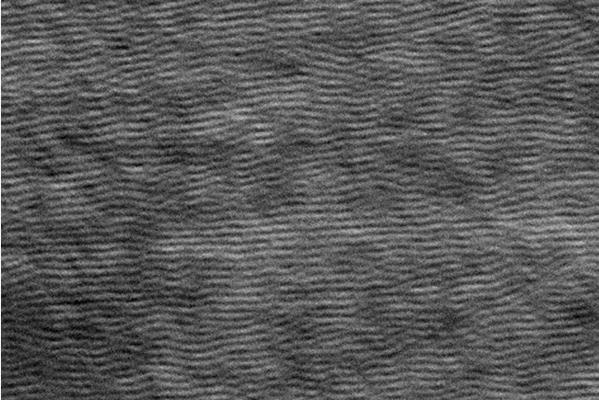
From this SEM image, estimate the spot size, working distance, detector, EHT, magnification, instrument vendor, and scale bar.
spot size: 4.6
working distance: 10.6 mm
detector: SE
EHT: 20 kV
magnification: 2 K X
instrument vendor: FEI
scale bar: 10000 nm